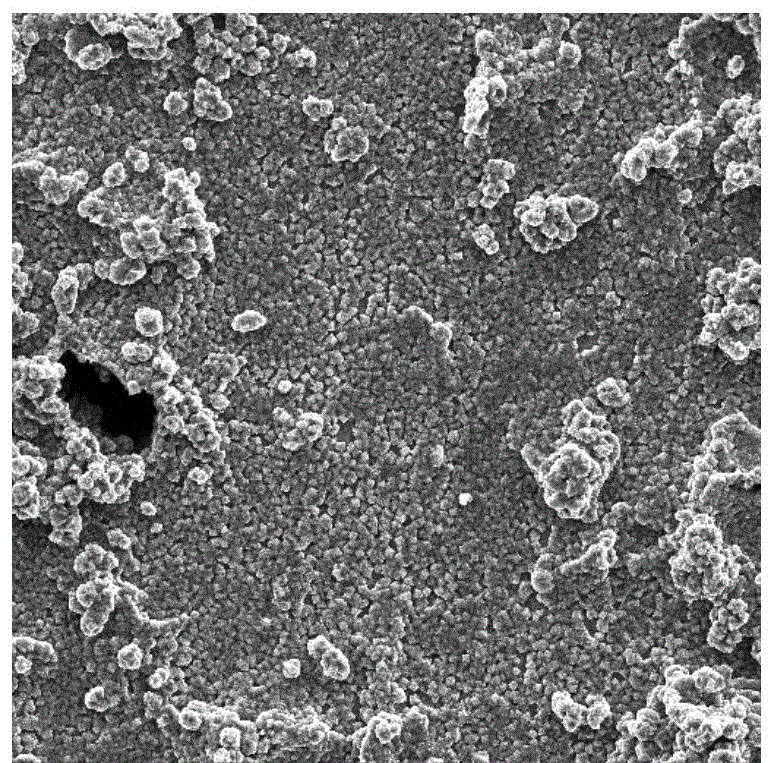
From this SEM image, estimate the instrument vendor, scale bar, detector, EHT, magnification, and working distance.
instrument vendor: Tescan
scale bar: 20000 nm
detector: SE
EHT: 15 kV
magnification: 1 K X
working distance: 4.97 mm